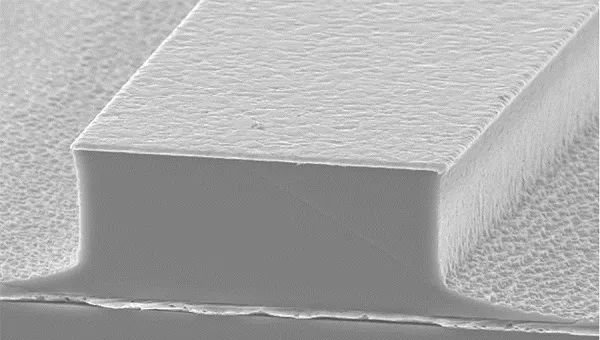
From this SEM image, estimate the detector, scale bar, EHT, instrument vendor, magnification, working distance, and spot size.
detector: SE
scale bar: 10000 nm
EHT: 15 kV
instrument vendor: FEI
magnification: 3.5 K X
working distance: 15.9 mm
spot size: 5